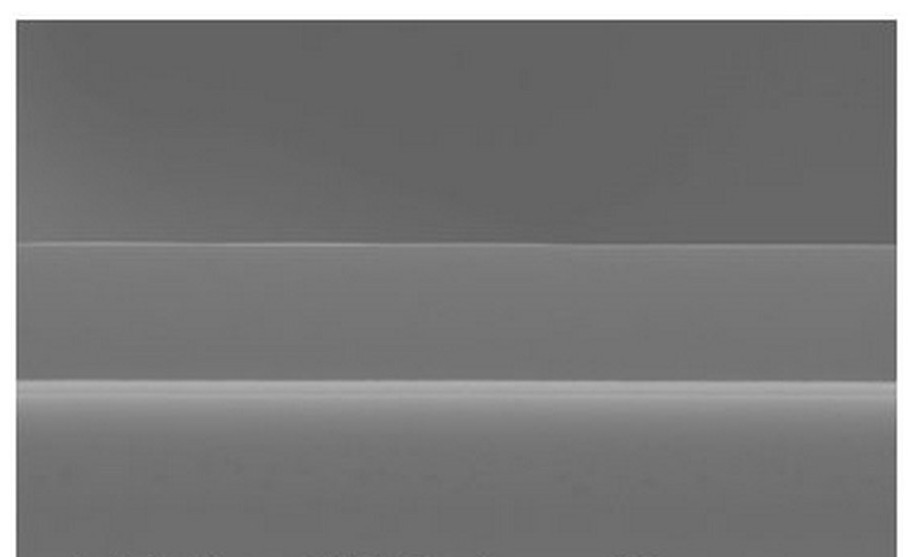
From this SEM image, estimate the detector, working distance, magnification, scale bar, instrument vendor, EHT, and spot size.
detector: SE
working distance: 10.6 mm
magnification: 9.495 K X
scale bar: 2000 nm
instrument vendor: FEI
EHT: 15 kV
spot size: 3